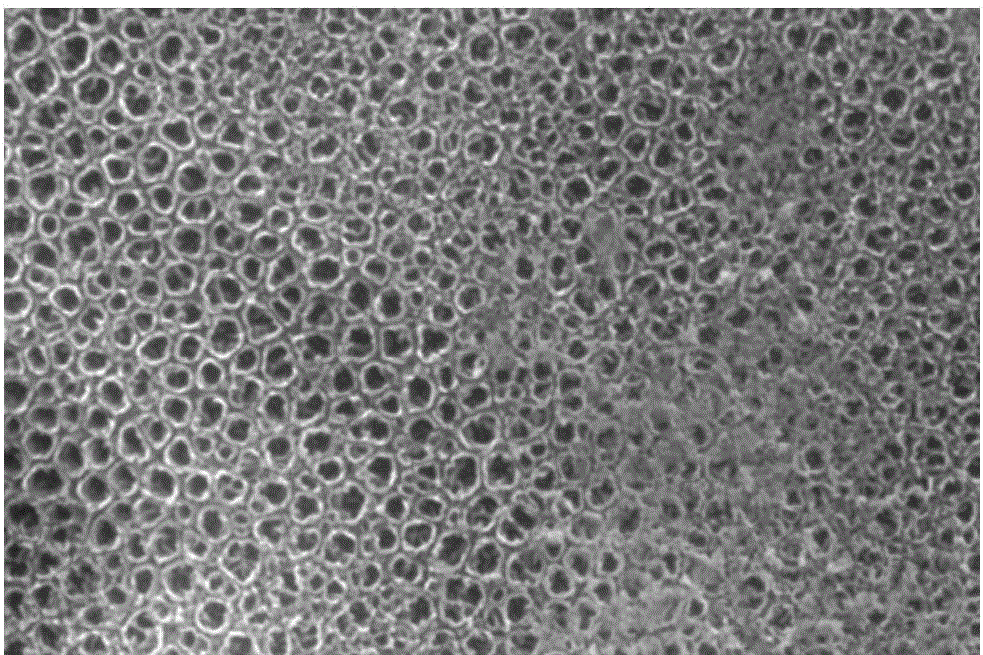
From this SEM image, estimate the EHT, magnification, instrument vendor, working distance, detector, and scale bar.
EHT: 15 kV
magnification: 100 K X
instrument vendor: FEI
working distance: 9.2 mm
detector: ETD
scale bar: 2000 nm